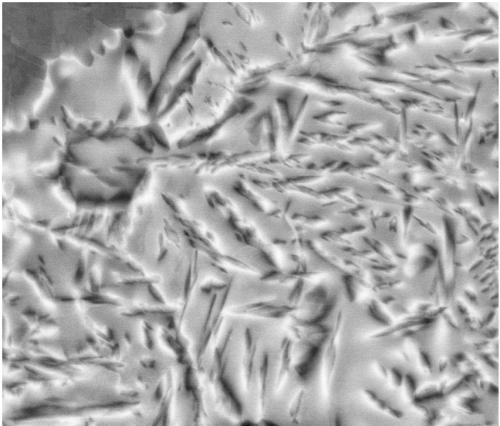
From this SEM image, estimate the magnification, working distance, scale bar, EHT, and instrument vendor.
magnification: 10 K X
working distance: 12.1 mm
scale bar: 10000 nm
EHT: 20 kV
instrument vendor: FEI